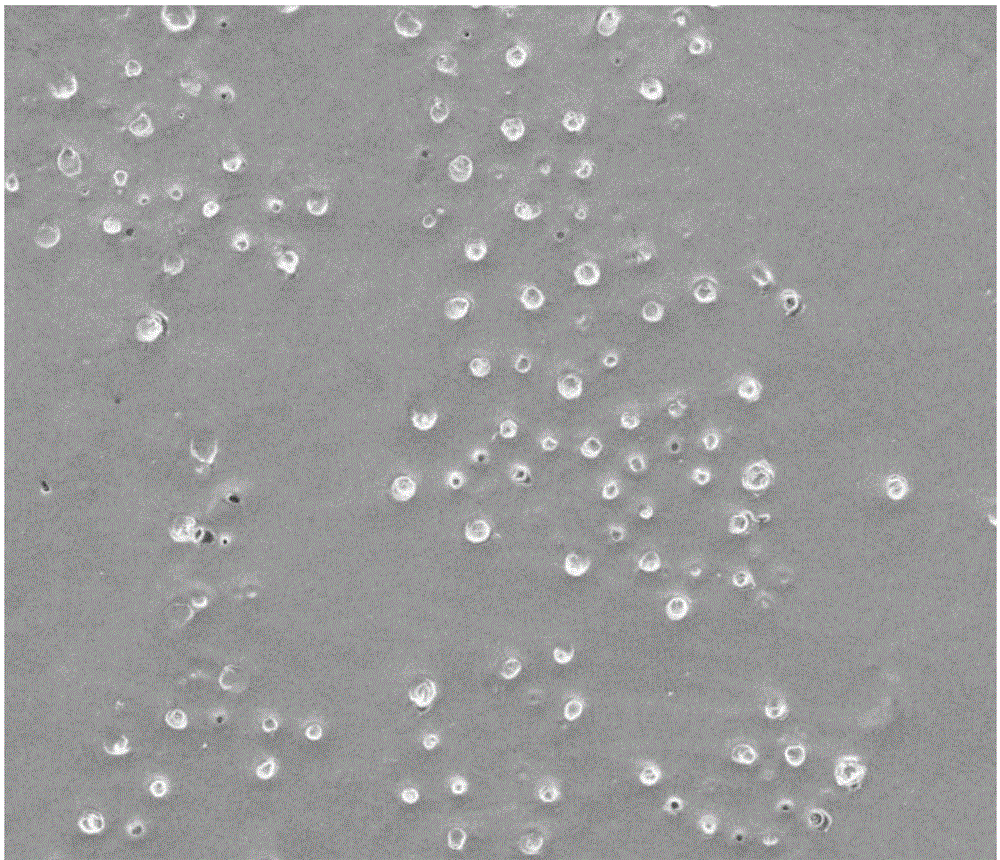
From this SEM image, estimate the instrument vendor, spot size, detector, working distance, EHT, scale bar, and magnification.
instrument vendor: FEI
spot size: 4.5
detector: ETD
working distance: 15.5 mm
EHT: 20 kV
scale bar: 500000 nm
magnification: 0.2 K X